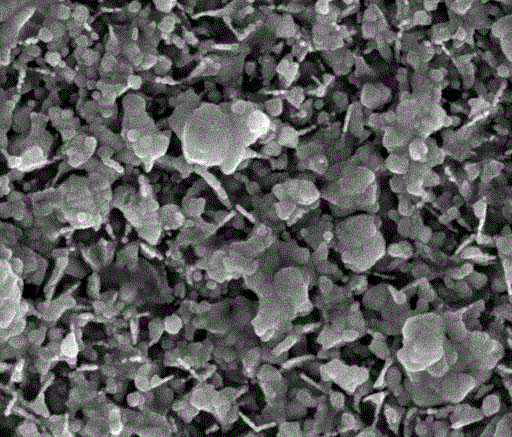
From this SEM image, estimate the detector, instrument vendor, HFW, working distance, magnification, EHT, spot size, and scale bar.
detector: ETD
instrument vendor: FEI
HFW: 2.98 µm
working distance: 6.7 mm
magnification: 50 K X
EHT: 10 kV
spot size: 2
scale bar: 500 nm